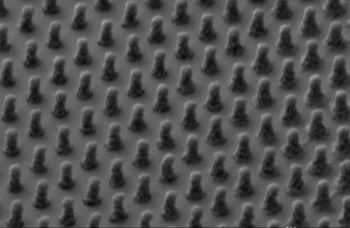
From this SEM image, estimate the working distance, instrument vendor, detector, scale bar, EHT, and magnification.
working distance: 5.6 mm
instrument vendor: Zeiss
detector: SE2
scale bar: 200 nm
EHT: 1 kV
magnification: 50 K X